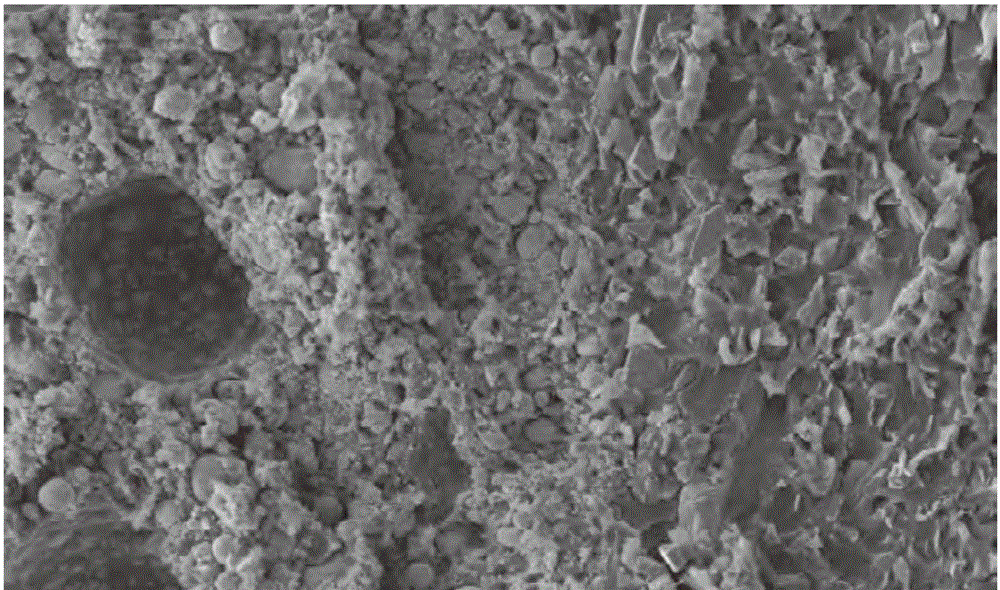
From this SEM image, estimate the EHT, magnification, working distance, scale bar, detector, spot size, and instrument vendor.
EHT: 20 kV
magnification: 0.5 K X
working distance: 6.4 mm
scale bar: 100000 nm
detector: SE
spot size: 4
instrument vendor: FEI